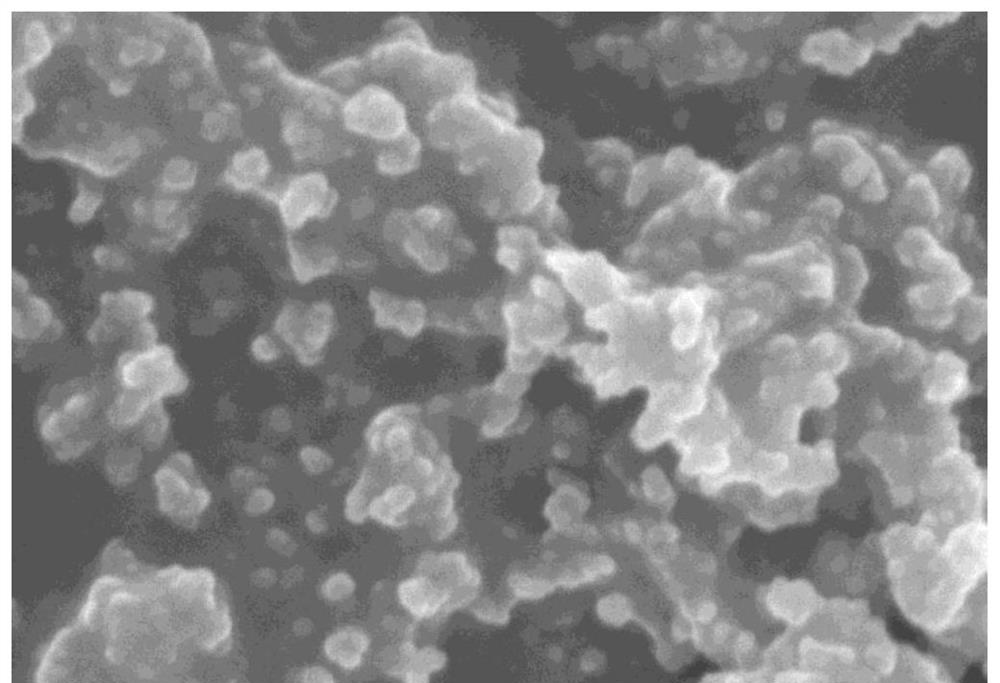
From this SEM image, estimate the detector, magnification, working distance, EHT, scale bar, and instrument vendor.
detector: SE(U)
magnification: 100 K X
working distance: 8.6 mm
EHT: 5 kV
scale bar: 500 nm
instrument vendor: Hitachi